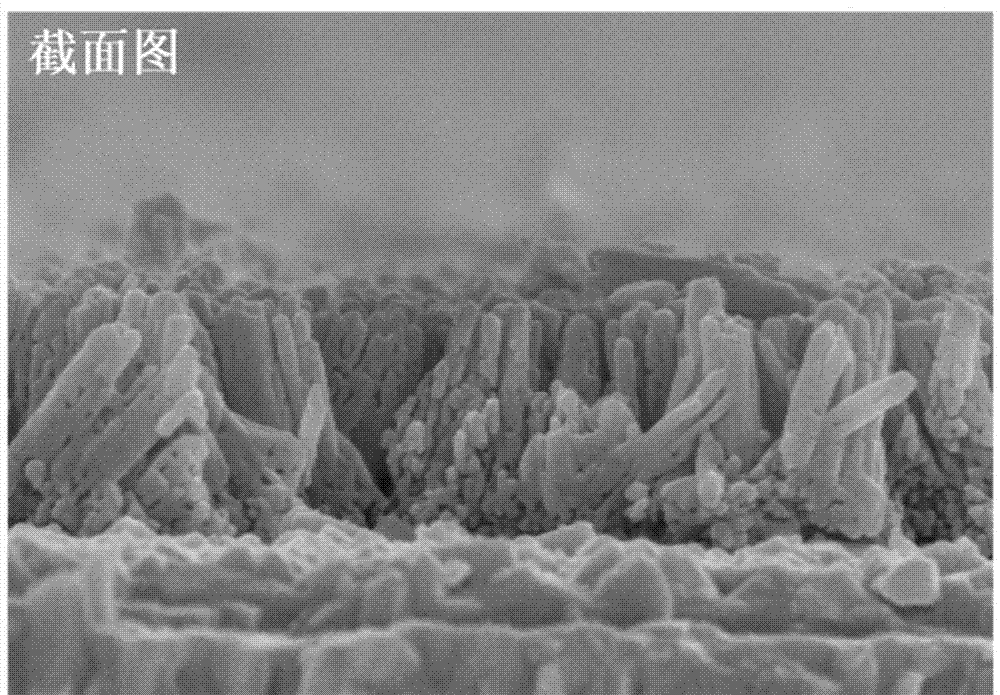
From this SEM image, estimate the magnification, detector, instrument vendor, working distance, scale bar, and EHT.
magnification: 80 K X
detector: SE(U)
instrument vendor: Hitachi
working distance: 8 mm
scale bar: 500 nm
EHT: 5 kV